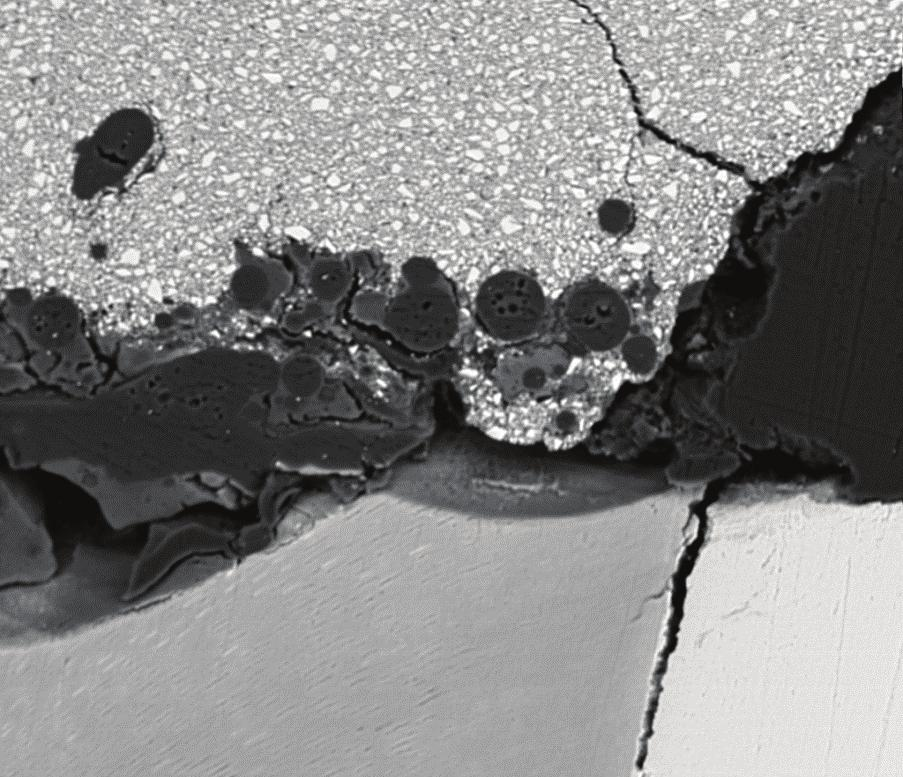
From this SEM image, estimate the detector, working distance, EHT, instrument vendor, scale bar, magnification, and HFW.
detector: SSD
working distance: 10 mm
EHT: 25 kV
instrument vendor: FEI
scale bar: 300000 nm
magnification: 0.149 K X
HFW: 860 µm